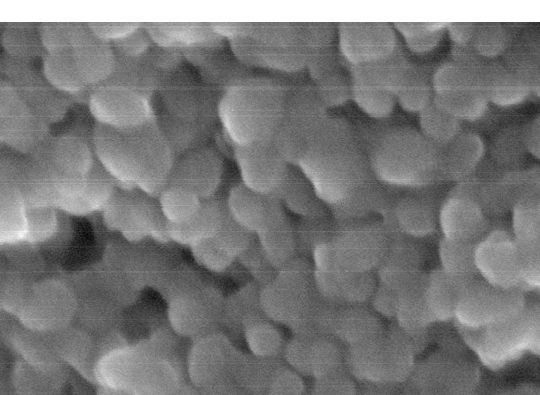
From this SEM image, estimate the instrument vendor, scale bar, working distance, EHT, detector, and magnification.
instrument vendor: Hitachi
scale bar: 100 nm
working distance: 8.3 mm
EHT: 30 kV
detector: SE(M)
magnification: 300 K X